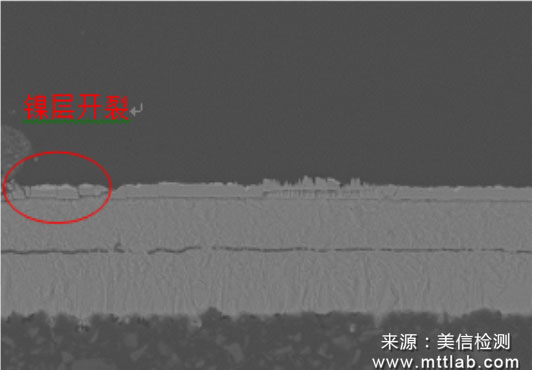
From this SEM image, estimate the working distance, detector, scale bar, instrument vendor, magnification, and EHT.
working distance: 10 mm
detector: BSE3D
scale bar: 50000 nm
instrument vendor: Hitachi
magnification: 1 K X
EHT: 15 kV